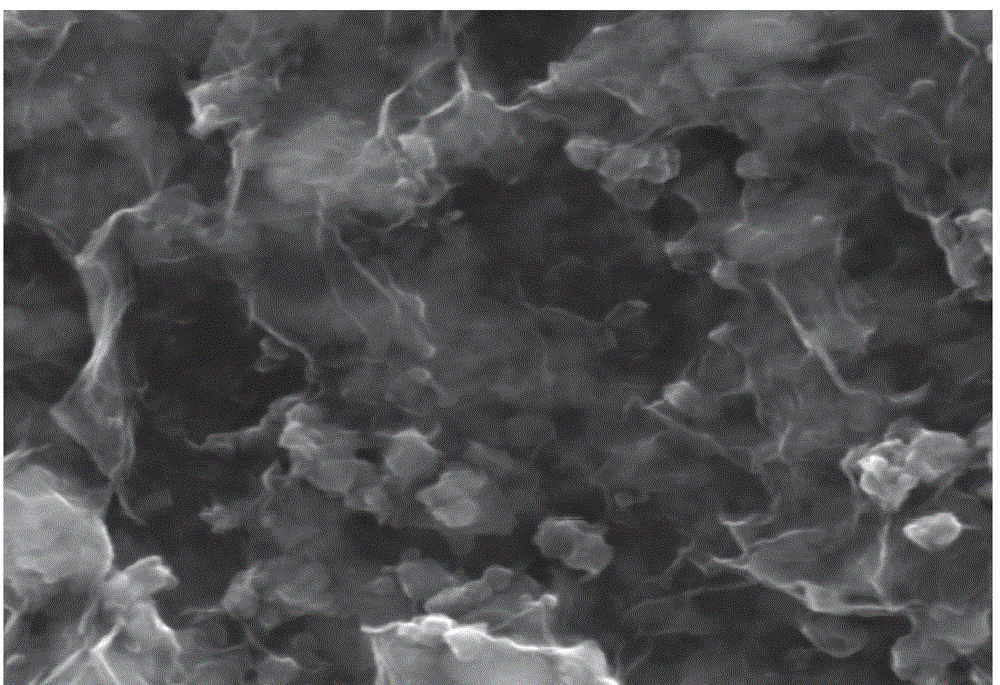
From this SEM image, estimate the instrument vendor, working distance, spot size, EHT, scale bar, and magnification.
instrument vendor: FEI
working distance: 9.9 mm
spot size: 3.5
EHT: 20 kV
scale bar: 1000 nm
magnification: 100 K X